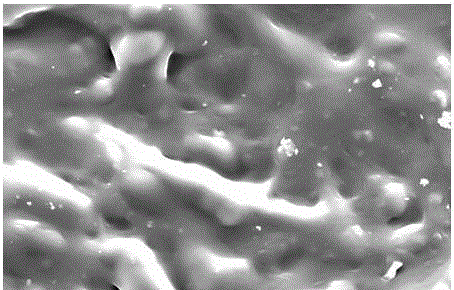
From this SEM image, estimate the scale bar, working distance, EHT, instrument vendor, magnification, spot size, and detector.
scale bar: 50000 nm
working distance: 15.3 mm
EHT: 20 kV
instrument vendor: FEI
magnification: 3 K X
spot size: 3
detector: ETD